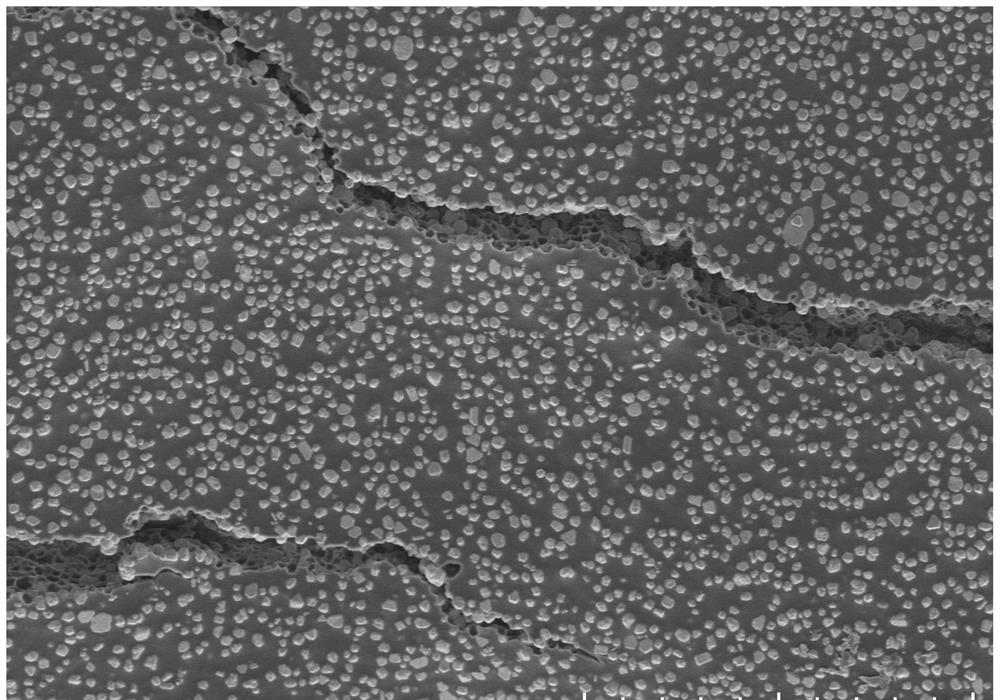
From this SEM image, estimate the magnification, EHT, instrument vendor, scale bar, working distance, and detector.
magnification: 1 K X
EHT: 5 kV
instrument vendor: Hitachi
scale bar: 50000 nm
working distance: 9.8 mm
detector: SE(UL)